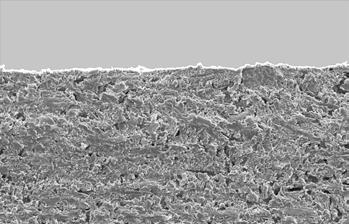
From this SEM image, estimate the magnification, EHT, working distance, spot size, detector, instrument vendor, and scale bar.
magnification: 0.1 K X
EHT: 16 kV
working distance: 12.2 mm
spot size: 5.2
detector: SE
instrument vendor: FEI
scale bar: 200000 nm